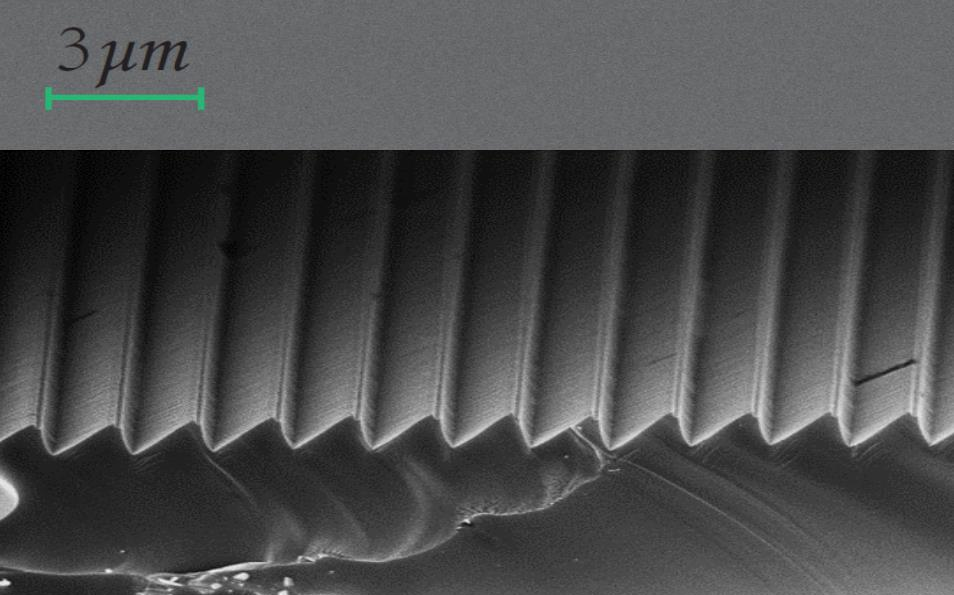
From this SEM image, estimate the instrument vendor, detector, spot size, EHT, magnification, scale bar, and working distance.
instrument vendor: FEI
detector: TLD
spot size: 4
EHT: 10 kV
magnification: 10 K X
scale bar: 5000 nm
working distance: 6.7 mm